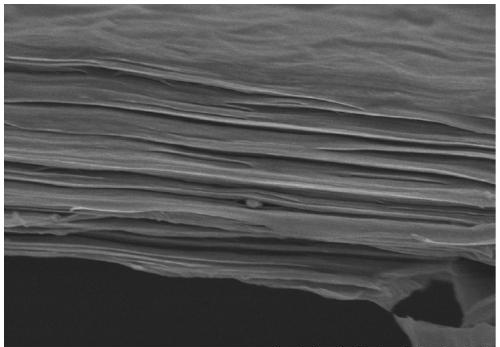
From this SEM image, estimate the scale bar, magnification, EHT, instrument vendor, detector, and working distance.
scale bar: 3000 nm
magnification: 18 K X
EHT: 3 kV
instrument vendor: Hitachi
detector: SE(M)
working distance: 10.5 mm